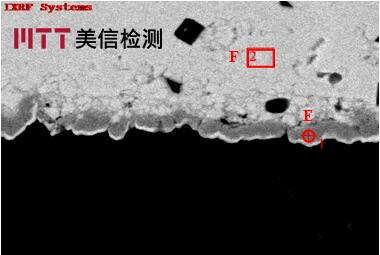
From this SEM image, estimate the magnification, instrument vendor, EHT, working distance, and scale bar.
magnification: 5 K X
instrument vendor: other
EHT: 15 kV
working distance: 10 mm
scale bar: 5000 nm